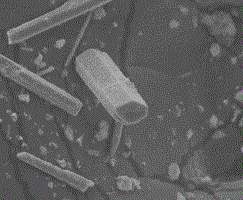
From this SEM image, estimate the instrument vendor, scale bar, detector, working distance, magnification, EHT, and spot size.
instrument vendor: FEI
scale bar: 50000 nm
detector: ETD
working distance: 9.9 mm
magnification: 1 K X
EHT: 20 kV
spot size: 3